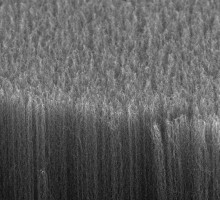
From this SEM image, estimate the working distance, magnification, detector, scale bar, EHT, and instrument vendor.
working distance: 15.9 mm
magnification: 2.5 K X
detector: SEI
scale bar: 10000 nm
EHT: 5 kV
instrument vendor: JEOL